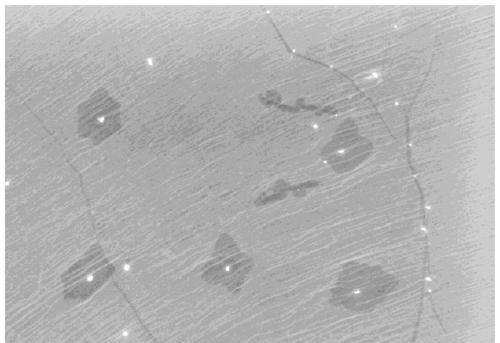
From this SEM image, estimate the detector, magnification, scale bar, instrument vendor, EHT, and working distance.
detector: SE(M)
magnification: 11 K X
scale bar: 5000 nm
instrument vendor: Hitachi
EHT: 5 kV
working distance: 8.3 mm